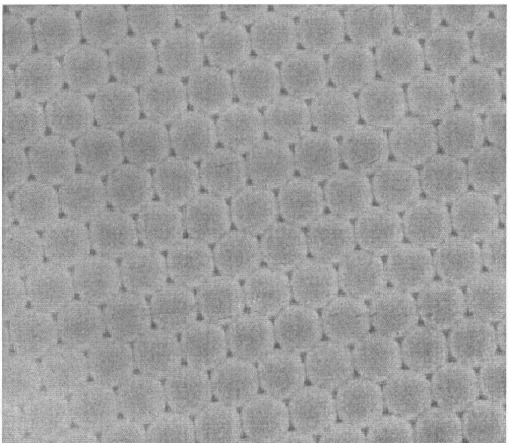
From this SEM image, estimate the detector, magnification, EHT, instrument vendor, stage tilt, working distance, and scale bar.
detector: LVSED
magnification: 60 K X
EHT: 15 kV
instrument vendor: FEI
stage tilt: -0°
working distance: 7.1 mm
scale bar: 1000 nm